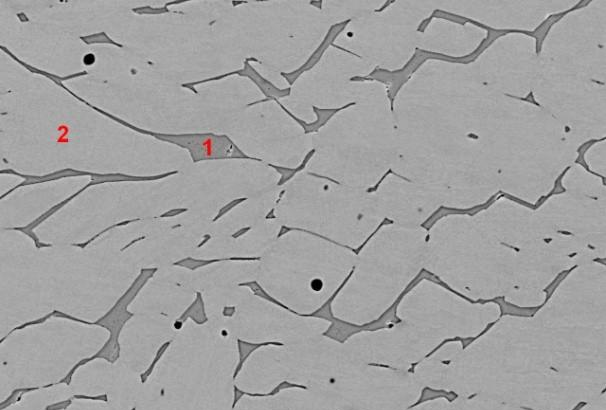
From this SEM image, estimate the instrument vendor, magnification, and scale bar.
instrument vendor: JEOL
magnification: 1 K X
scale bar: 10000 nm